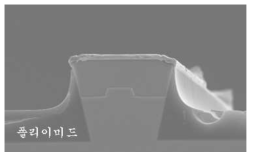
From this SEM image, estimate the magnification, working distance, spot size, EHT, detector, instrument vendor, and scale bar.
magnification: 5 K X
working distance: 8.4 mm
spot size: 3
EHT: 10 kV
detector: SE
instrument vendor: FEI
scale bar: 5000 nm